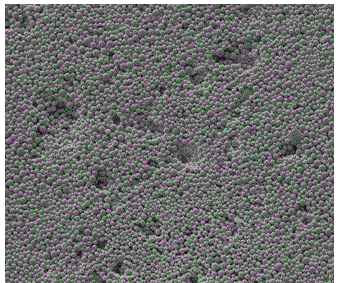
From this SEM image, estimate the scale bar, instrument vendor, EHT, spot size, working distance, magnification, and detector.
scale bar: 10000 nm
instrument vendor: FEI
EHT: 5 kV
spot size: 3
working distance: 6.2 mm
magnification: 5 K X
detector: ETD